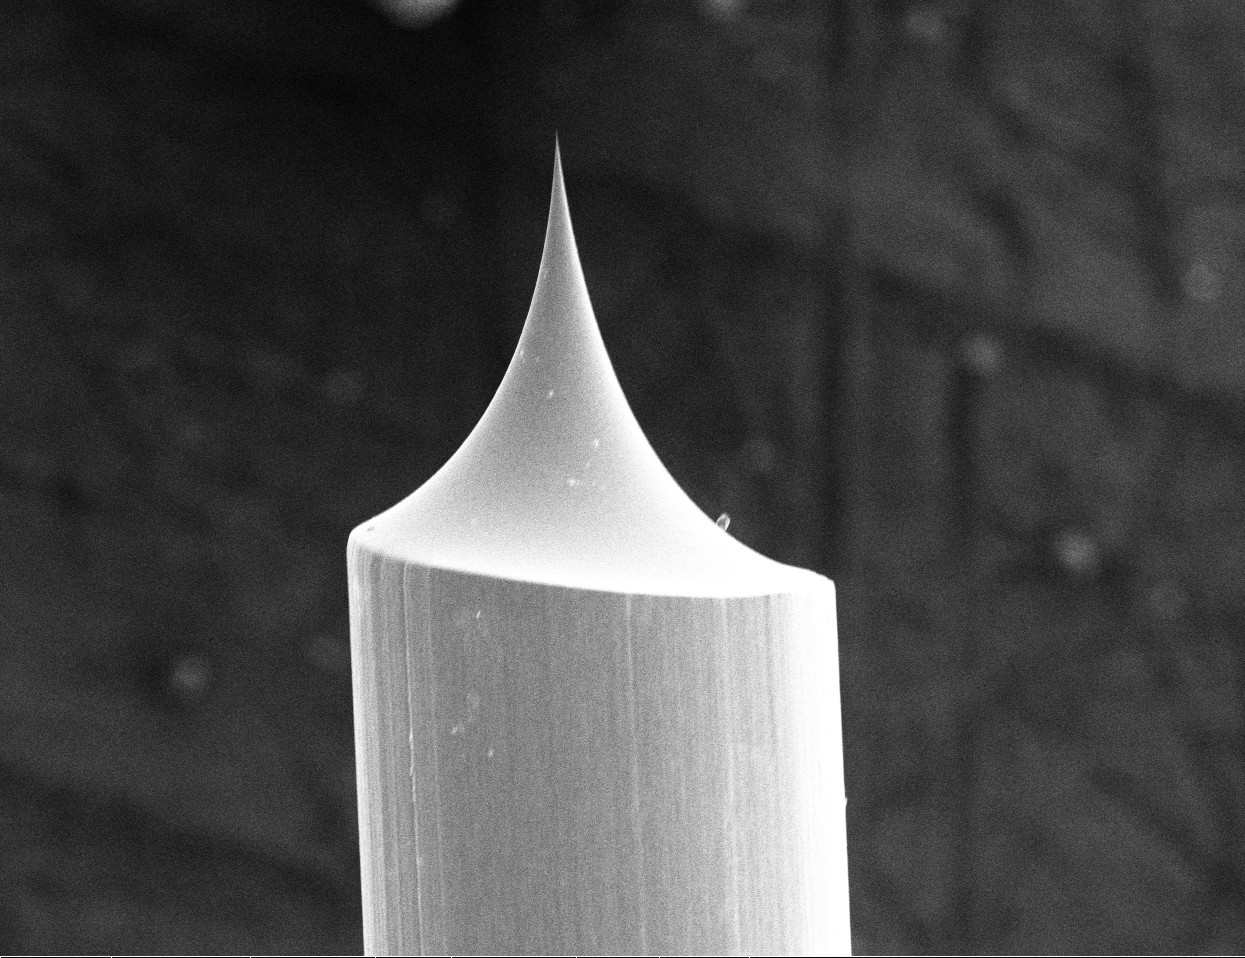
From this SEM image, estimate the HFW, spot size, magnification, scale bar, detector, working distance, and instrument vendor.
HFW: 834 µm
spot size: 4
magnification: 0.497 K X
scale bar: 200000 nm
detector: ETD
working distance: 20.5 mm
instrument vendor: FEI